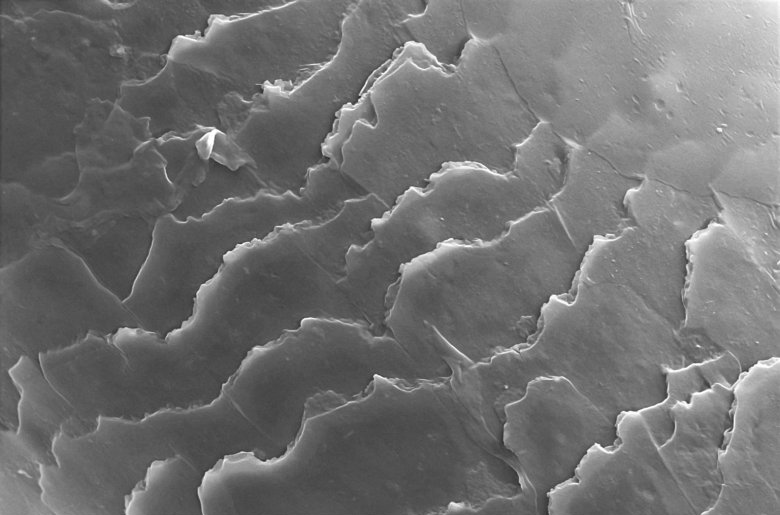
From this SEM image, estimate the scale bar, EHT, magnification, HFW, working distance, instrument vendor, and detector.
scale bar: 10000 nm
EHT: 15 kV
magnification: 2 K X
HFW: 63.5 µm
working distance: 8.1 mm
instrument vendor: Thermo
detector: ETD